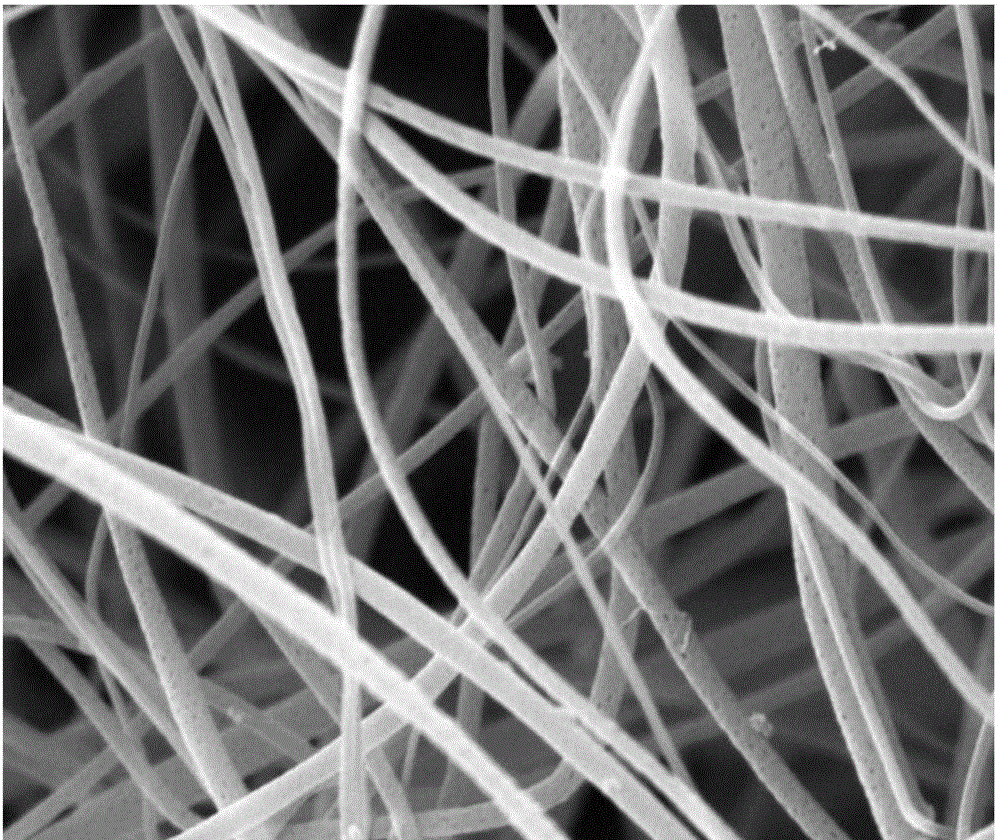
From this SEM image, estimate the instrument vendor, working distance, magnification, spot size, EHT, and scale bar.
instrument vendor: FEI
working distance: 10.9 mm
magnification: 20 K X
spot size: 3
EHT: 20 kV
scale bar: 5000 nm